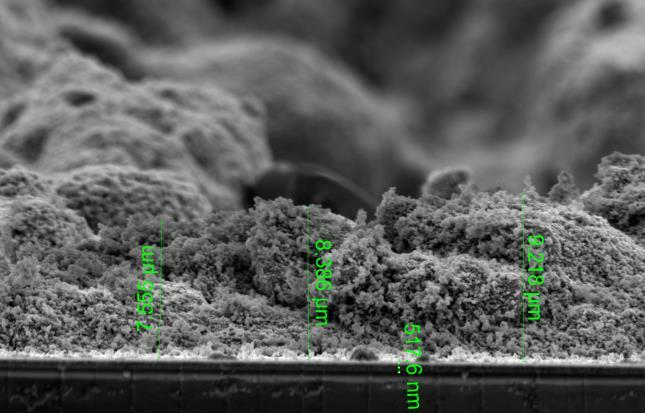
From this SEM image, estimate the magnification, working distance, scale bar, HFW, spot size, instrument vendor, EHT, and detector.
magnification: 12 K X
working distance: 10.1 mm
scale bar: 10000 nm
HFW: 34.5 µm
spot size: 3.5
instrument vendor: FEI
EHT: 5 kV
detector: ETD